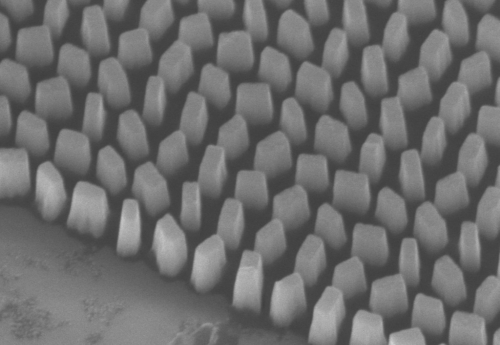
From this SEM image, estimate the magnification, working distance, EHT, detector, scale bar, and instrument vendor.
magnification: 40 K X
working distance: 12.1 mm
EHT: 5 kV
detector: SE(U)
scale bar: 1000 nm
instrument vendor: Hitachi